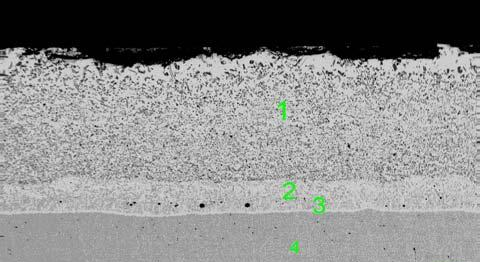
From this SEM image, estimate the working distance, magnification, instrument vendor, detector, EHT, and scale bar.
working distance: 11 mm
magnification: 0.1 K X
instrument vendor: Zeiss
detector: CZ BSD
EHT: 20 kV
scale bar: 100000 nm